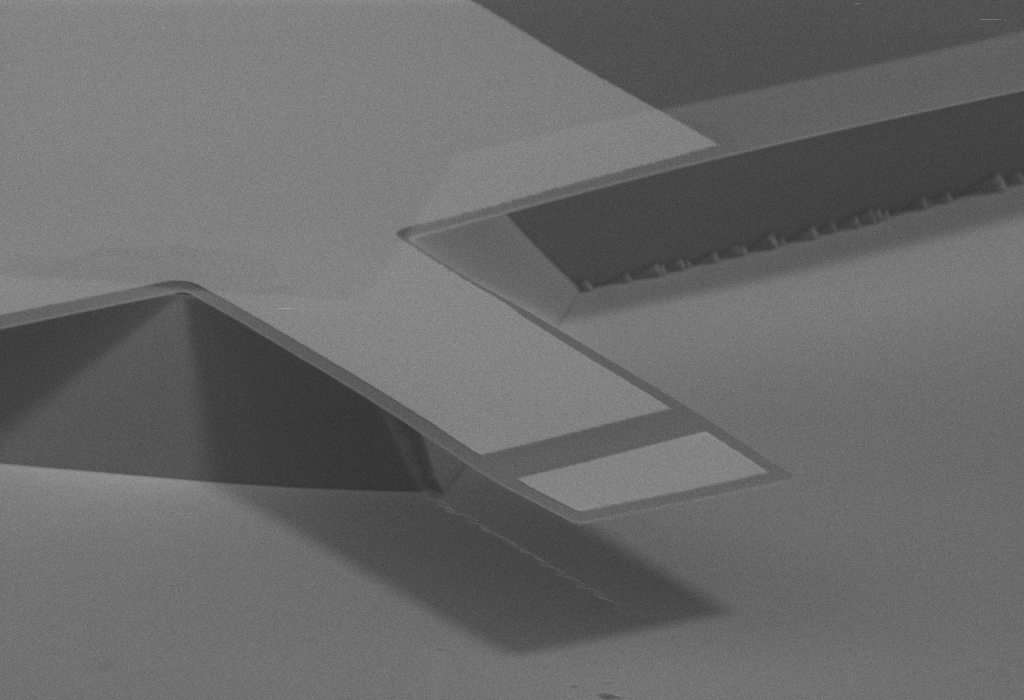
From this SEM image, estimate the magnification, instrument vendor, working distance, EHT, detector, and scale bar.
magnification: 1.63 K X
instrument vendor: Zeiss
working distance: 35 mm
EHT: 13 kV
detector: MPSE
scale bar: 20000 nm